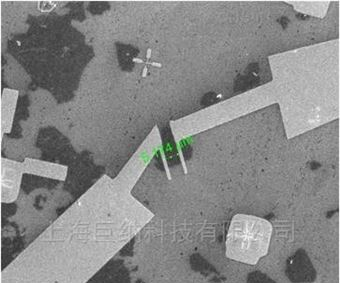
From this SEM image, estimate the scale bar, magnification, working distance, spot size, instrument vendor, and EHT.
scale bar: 50000 nm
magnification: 2.5 K X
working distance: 8.6 mm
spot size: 3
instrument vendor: FEI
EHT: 20 kV